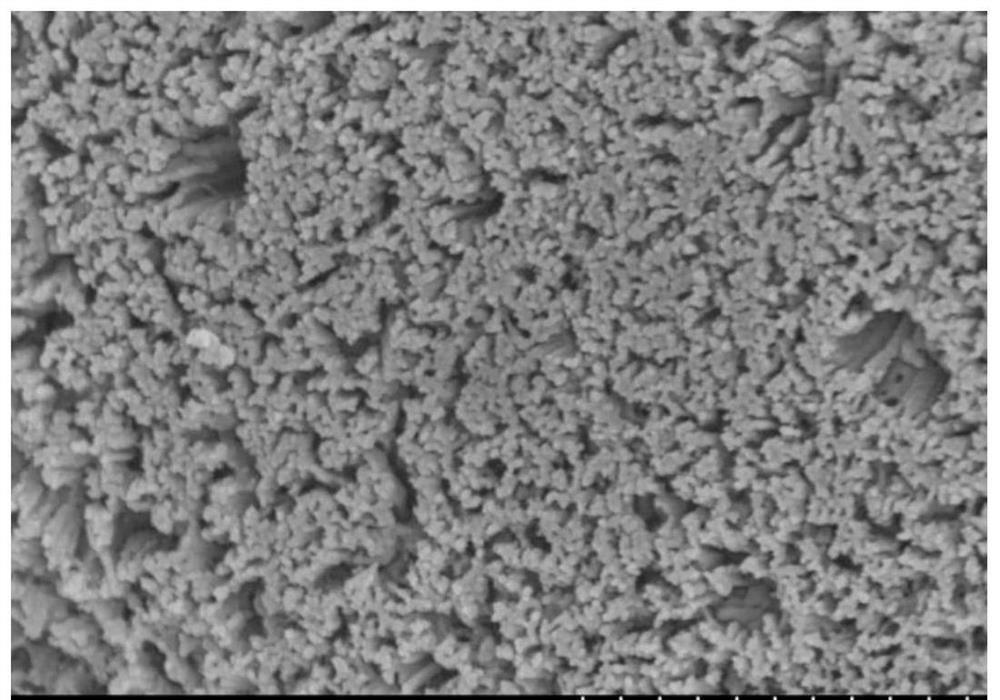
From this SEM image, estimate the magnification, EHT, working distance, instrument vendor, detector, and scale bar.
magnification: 50 K X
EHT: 5 kV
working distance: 8.4 mm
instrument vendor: Hitachi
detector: SE(M)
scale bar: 1000 nm